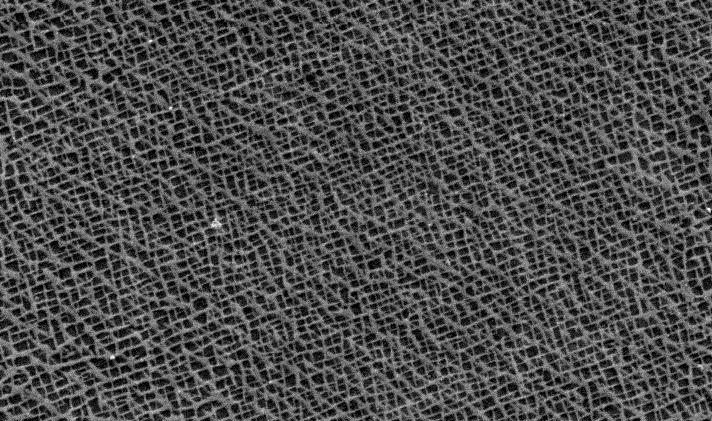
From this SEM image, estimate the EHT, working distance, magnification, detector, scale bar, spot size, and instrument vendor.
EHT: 20 kV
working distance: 10.6 mm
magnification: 2.5 K X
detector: SE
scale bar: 10000 nm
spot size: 4.4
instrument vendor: FEI